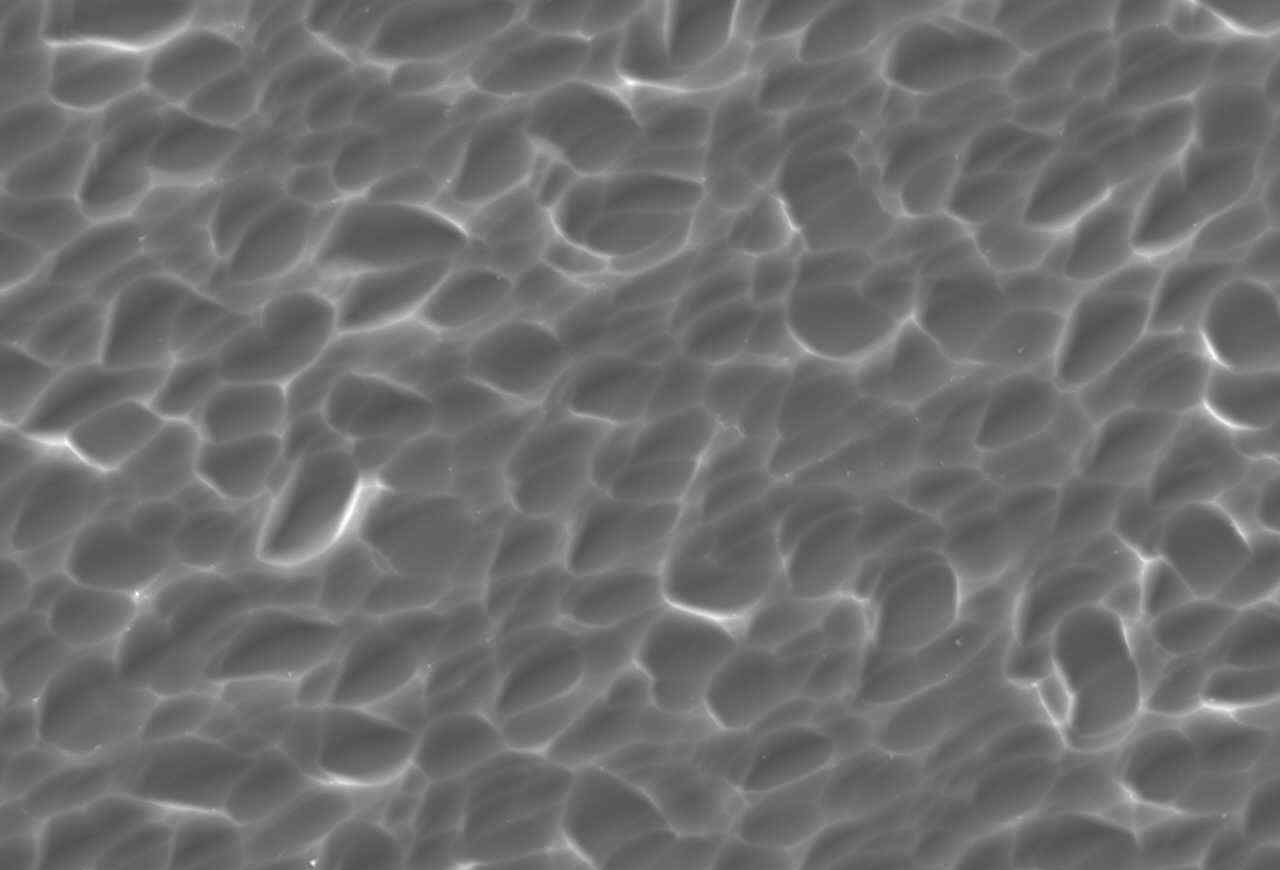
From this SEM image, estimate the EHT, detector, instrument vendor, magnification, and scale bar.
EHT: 20 kV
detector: SEI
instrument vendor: JEOL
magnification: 1 K X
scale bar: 10000 nm